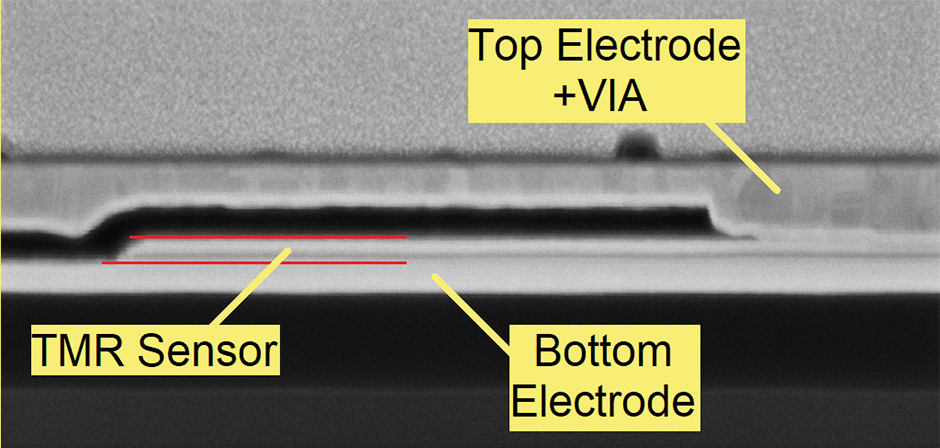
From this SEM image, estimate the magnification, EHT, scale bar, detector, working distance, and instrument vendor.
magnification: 50 K X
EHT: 5 kV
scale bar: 200 nm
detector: InLens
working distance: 5 mm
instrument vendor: Zeiss